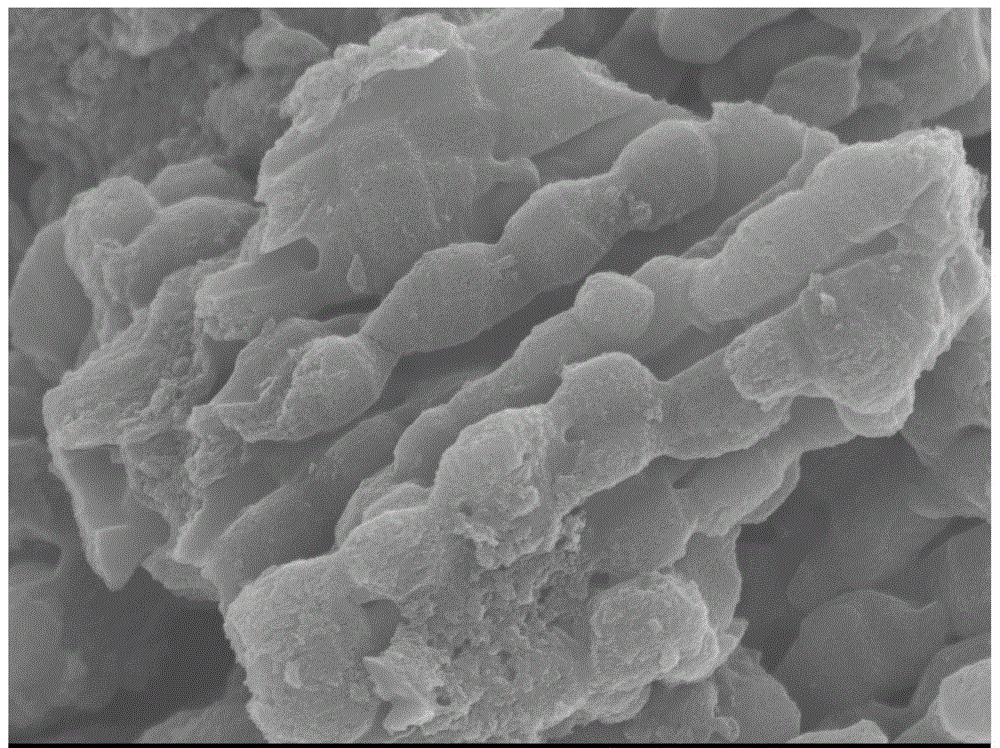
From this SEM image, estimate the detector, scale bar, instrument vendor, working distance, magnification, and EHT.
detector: SEI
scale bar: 1000 nm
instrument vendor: JEOL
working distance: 8.7 mm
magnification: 20 K X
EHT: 5 kV